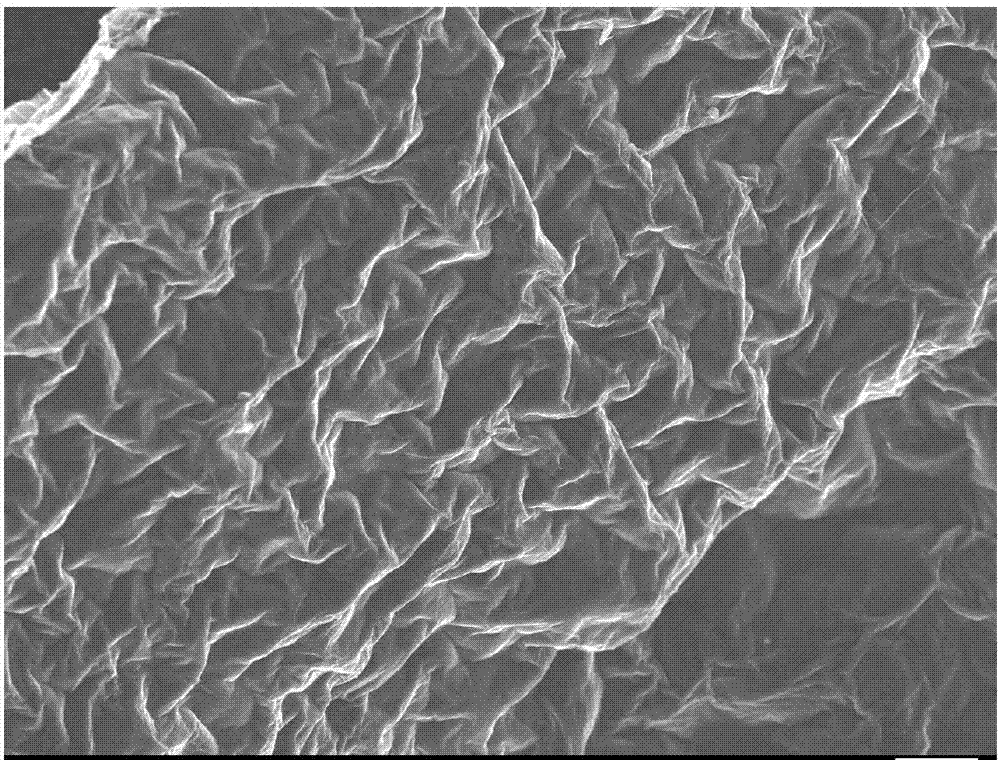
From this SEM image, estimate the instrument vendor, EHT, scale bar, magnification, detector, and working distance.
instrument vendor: JEOL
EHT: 10 kV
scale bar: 10000 nm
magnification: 1 K X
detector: SEI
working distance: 8.4 mm